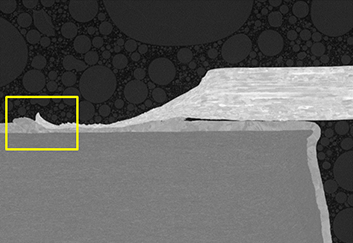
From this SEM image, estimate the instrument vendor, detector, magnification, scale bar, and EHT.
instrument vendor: Hitachi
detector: BSE-COMP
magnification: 1 K X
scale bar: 50000 nm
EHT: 5 kV